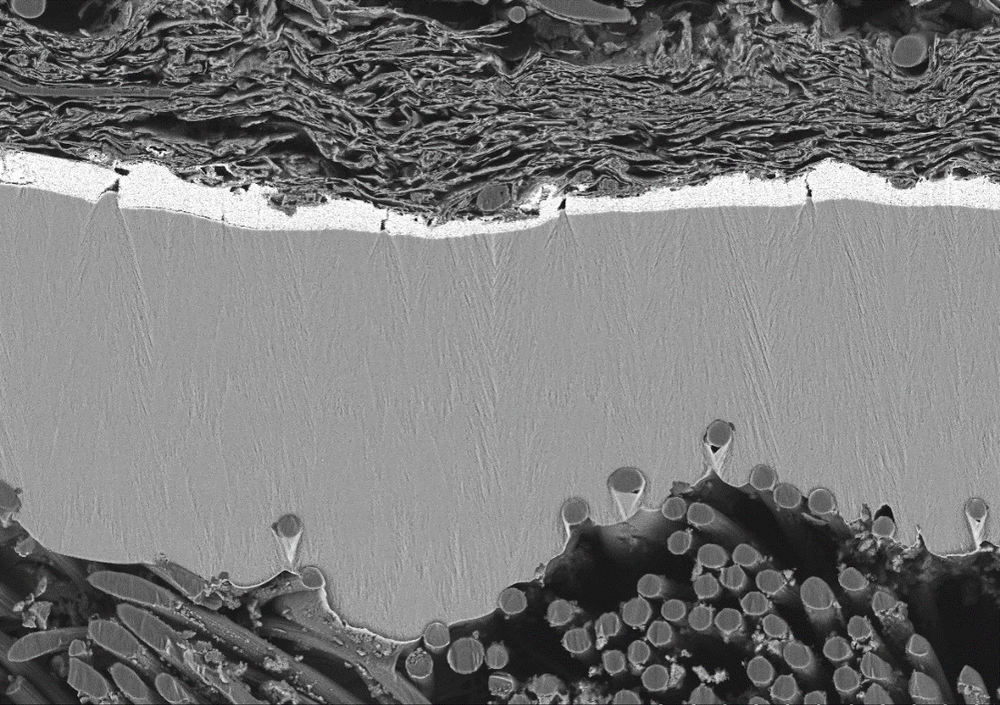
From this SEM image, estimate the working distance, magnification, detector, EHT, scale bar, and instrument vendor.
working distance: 5.4 mm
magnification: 0.4 K X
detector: BSE-COMP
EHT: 10 kV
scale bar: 100000 nm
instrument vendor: Hitachi